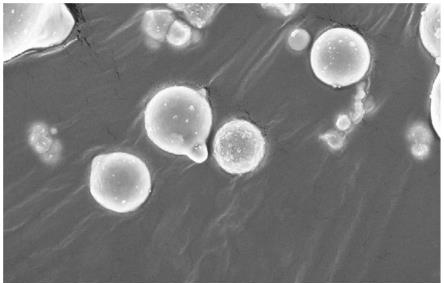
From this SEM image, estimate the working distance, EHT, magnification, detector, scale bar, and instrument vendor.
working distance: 10.7 mm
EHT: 9 kV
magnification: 20 K X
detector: InLens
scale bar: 1000 nm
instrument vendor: Zeiss